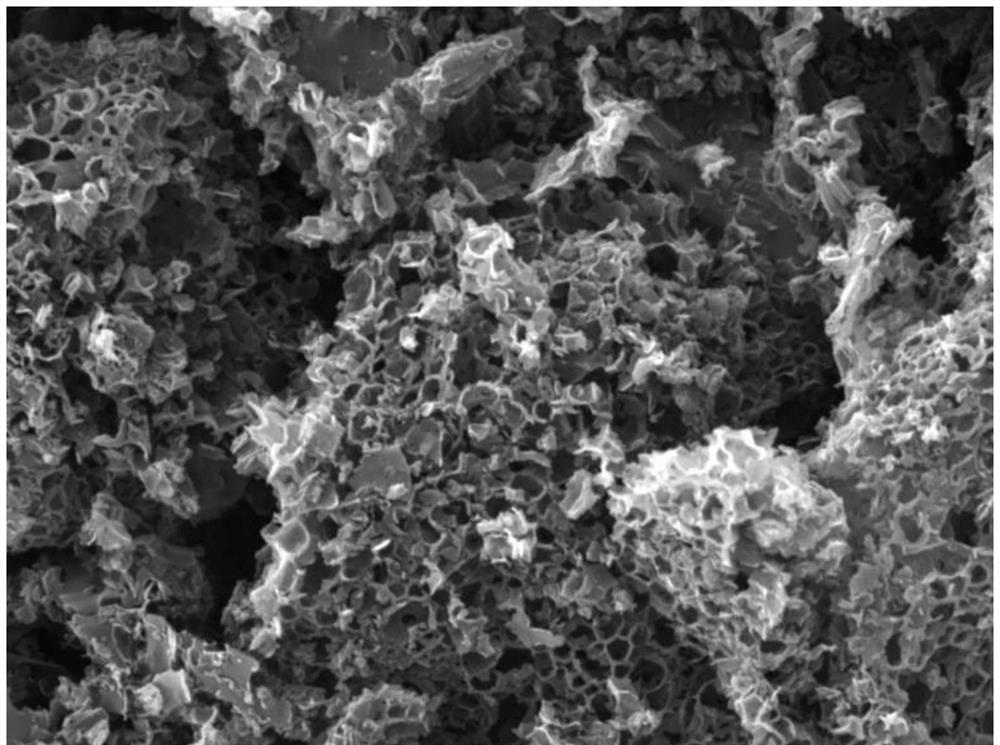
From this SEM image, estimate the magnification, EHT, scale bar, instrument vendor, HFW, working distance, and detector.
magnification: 10 K X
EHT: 20 kV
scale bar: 5000 nm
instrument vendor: Tescan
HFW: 19 µm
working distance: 8.75 mm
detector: SE